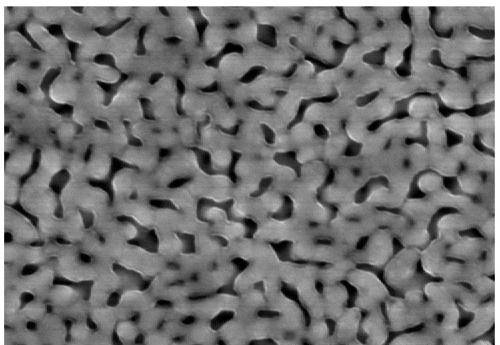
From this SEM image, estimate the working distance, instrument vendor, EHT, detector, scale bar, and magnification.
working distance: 11.3 mm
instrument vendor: Hitachi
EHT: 15 kV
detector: SE(U)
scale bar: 500 nm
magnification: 100 K X